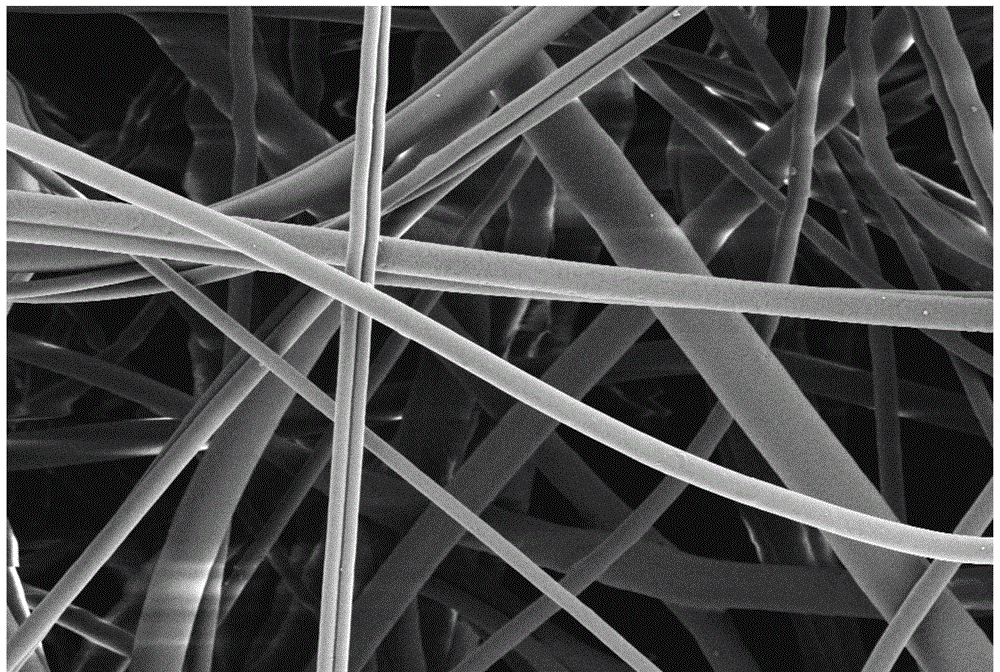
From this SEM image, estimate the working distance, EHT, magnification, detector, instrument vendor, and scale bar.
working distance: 4.8 mm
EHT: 2 kV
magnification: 2 K X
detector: SE2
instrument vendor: Zeiss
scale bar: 2000 nm